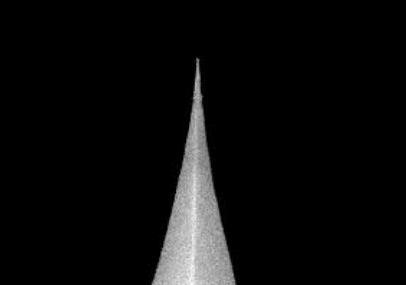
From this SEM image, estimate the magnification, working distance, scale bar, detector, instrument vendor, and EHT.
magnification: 50 K X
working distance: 7.2 mm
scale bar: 100 nm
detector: SEI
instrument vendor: JEOL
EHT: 20 kV